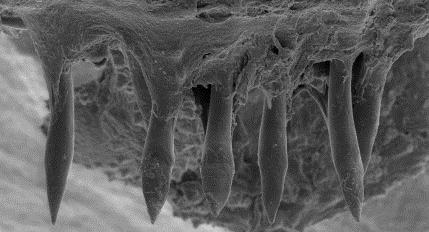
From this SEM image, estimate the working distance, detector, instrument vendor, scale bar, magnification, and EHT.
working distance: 9 mm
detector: SE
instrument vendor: other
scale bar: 100000 nm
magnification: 0.155 K X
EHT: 15 kV